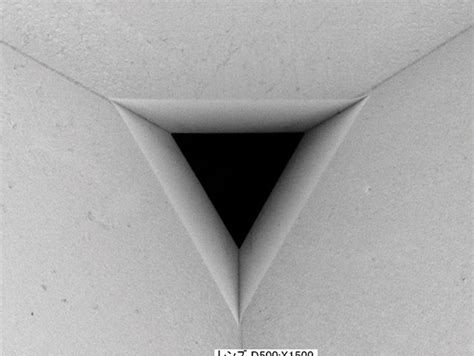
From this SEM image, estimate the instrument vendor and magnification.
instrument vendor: Keyence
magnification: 1.5 K X